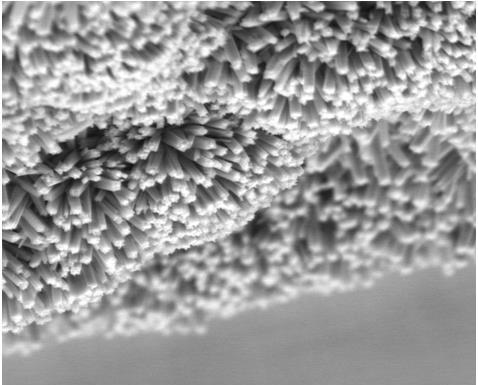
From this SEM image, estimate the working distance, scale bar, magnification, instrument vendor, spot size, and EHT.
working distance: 14.7 mm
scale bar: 5000 nm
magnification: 12 K X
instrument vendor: FEI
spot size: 3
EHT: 8 kV